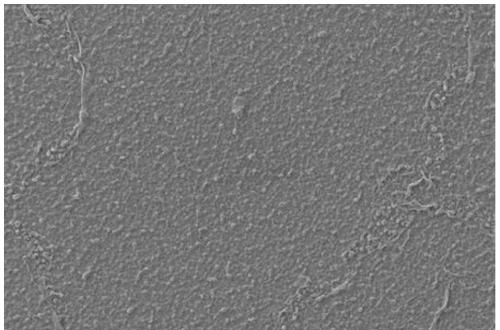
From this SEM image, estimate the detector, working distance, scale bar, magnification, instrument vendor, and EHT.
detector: SE1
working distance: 8.5 mm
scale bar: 1000 nm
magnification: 5 K X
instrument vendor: Zeiss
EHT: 20 kV